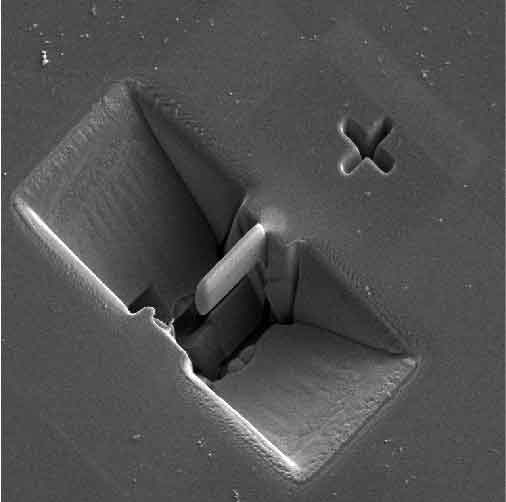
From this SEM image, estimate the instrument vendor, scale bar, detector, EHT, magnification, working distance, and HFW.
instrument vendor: Tescan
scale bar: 10000 nm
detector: SE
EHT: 5 kV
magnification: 6.33 K X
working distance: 9 mm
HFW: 32.8 µm